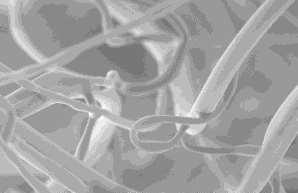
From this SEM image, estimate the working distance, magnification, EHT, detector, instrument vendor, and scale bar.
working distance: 9 mm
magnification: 0.5 K X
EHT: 5 kV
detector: SE2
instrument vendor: Zeiss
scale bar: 100000 nm